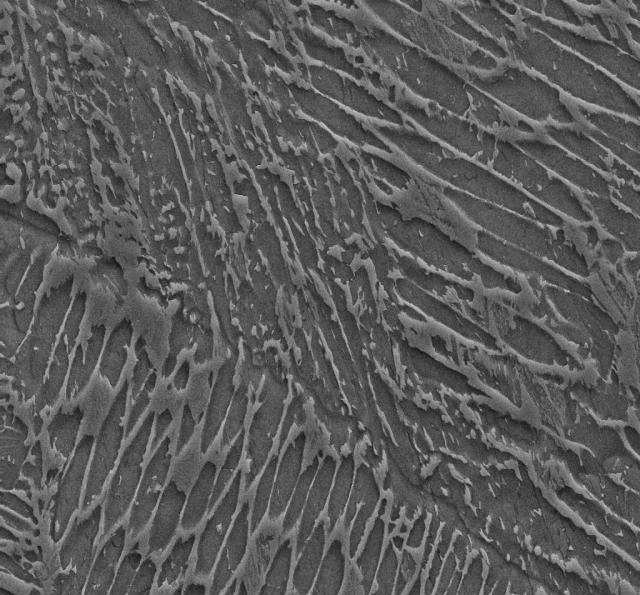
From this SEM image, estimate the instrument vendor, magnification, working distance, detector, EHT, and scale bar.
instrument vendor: Zeiss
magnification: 10 K X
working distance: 8 mm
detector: SE2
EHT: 15 kV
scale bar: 2000 nm